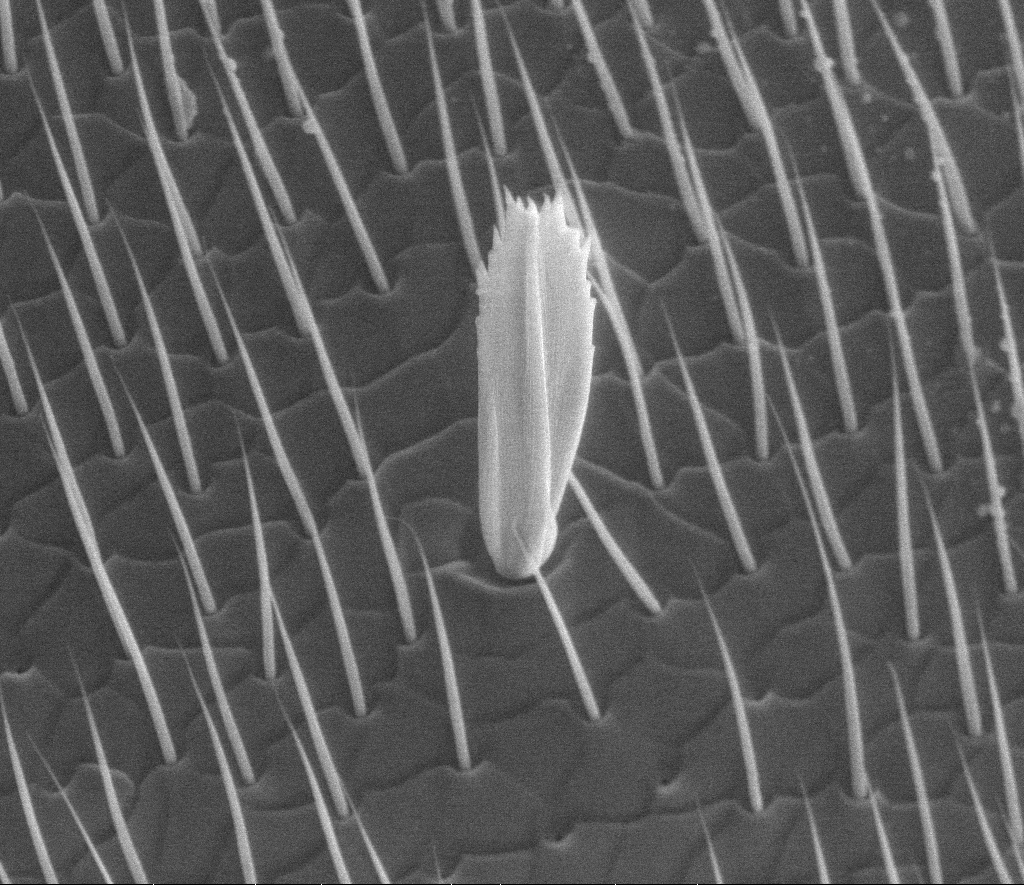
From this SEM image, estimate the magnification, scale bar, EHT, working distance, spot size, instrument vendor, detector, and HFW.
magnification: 2.221 K X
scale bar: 20000 nm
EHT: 10 kV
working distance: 11.06 mm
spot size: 3.5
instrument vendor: FEI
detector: SE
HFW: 120 µm